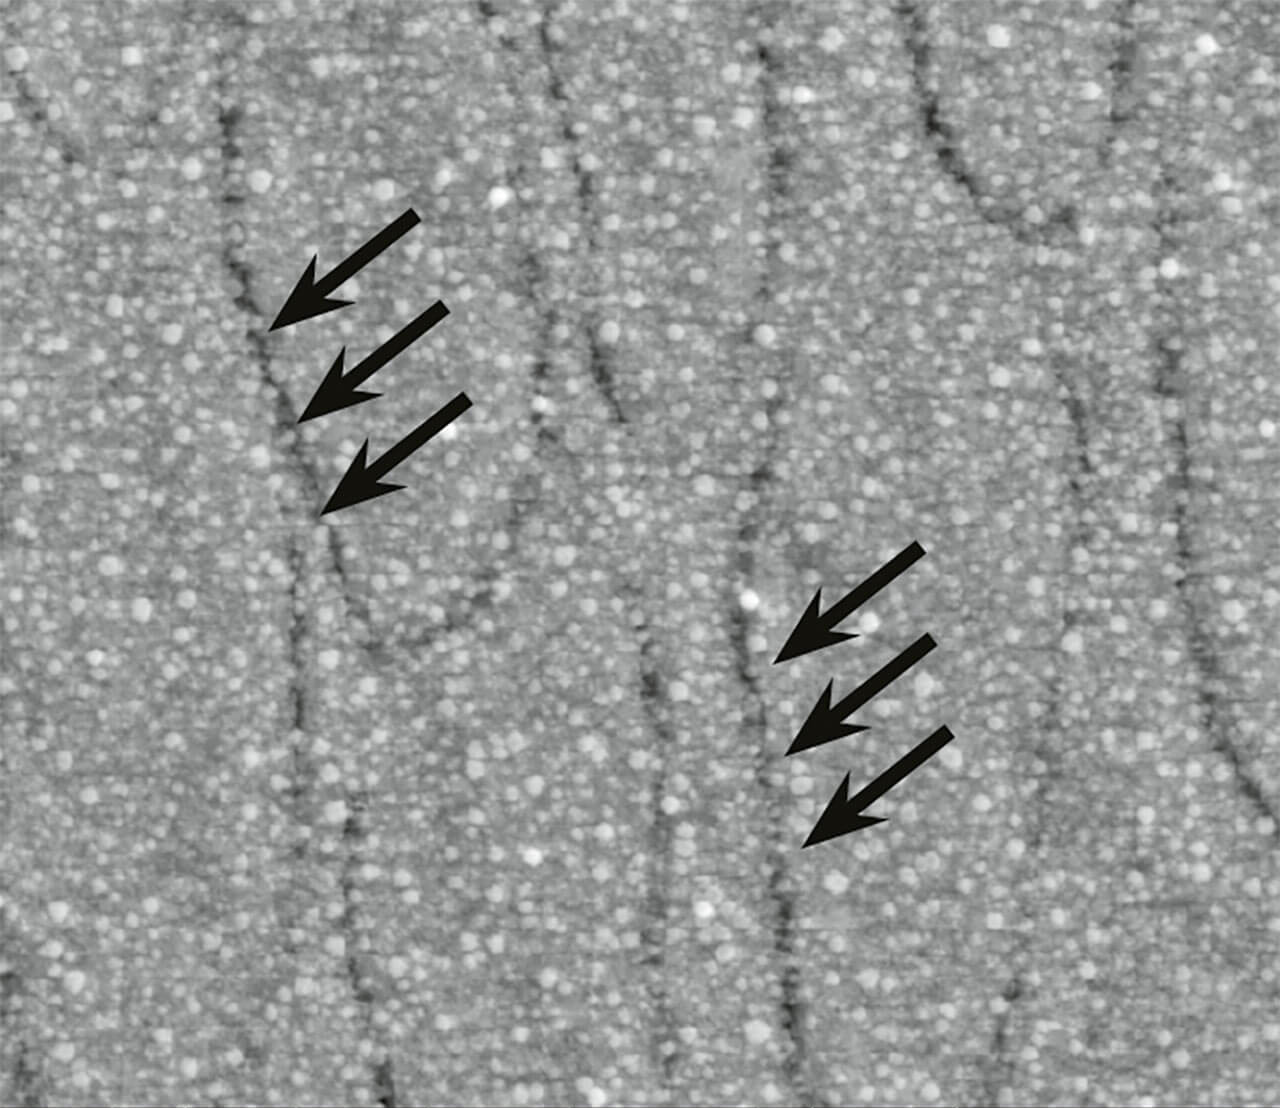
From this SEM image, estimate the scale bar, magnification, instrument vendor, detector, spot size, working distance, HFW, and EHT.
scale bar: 2000 nm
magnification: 25 K X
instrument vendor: FEI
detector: SE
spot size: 4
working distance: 10 mm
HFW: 10.82 µm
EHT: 10 kV